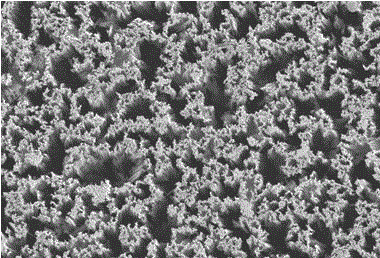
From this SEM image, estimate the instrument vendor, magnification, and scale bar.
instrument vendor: JEOL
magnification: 5 K X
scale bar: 5000 nm